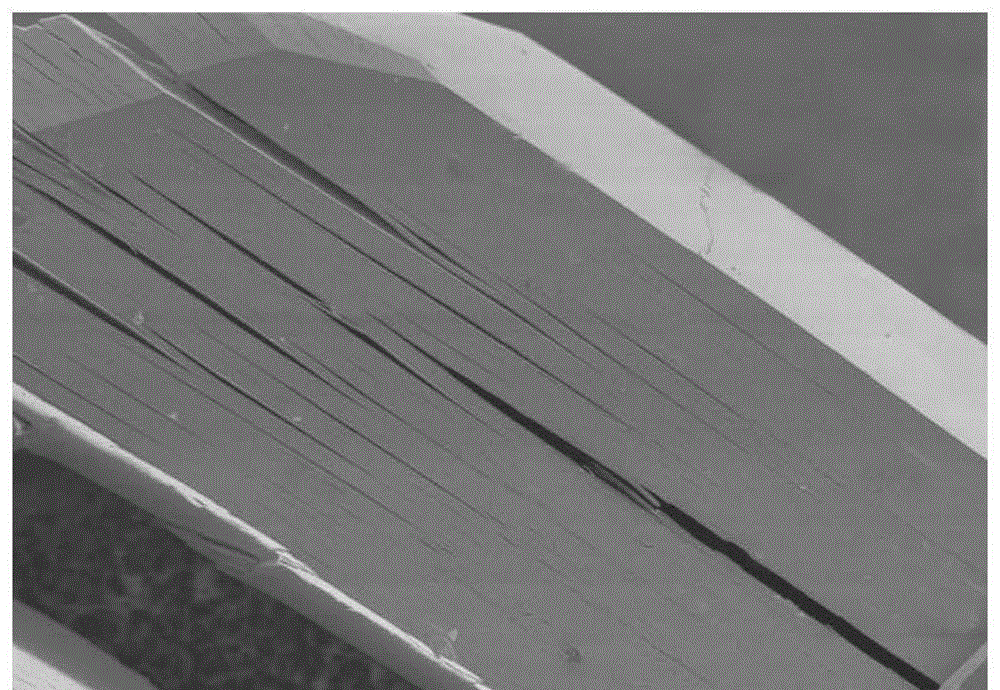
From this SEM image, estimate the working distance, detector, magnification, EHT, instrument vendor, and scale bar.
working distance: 8.1 mm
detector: SE(U)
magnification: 0.8 K X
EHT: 2 kV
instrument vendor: Hitachi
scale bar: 50000 nm